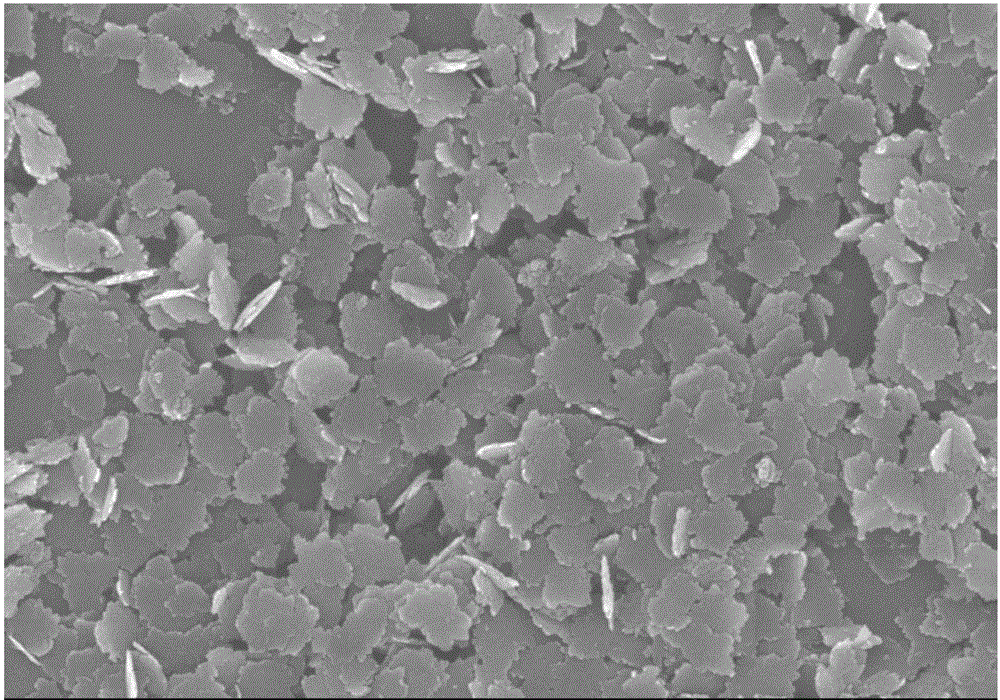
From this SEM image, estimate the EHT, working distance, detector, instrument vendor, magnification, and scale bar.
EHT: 15 kV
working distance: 14.9 mm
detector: SE(M)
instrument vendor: Hitachi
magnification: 20 K X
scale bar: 2000 nm